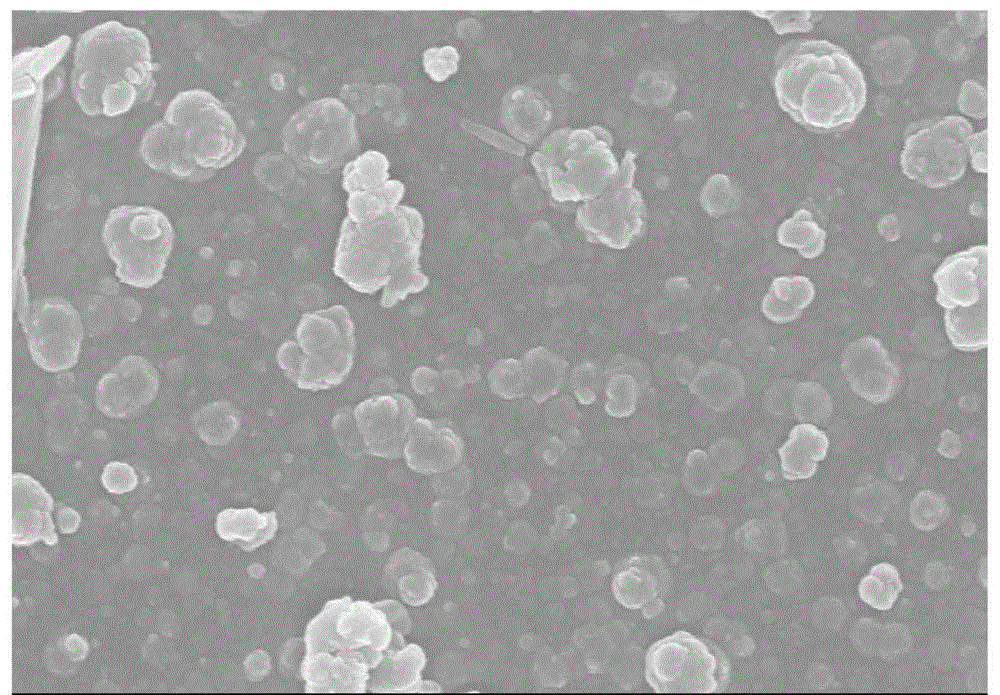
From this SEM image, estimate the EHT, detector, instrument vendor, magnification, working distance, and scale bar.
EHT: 15 kV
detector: SE(M)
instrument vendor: Hitachi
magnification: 20 K X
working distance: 11 mm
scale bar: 2000 nm